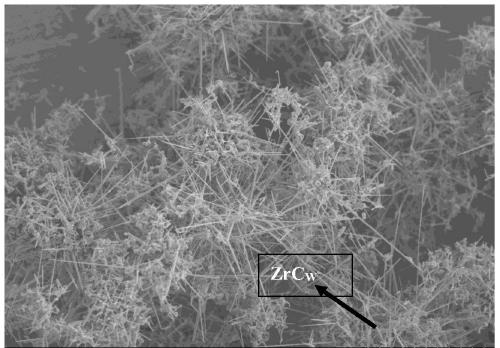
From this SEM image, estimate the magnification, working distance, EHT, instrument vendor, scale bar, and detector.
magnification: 0.3 K X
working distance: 14.3 mm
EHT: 15 kV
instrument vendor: Hitachi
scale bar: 100000 nm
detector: SE(M)